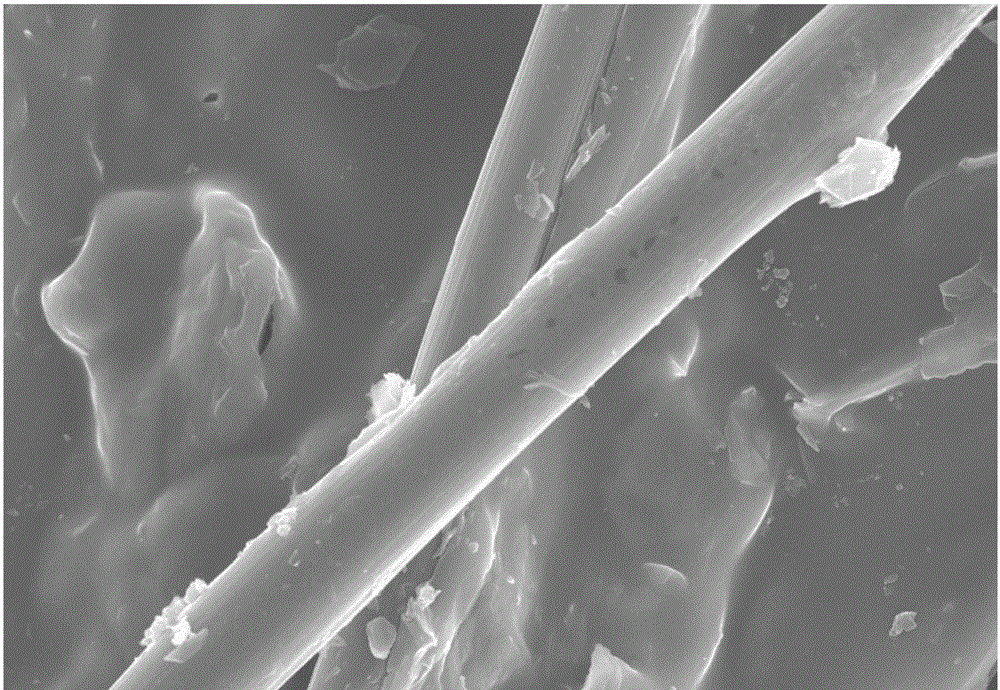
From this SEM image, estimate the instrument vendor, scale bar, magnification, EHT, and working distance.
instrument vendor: Hitachi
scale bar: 30000 nm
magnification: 1.5 K X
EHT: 20 kV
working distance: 11.8 mm